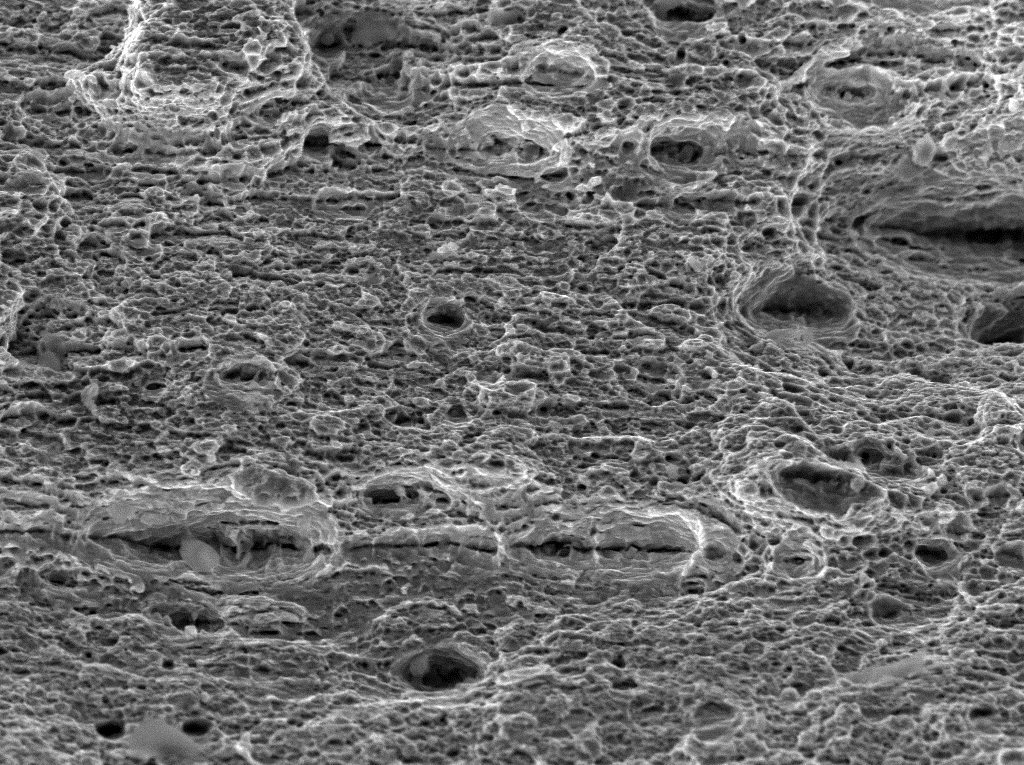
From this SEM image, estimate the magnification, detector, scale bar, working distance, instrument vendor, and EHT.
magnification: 1 K X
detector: SE Detector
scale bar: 50000 nm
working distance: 17.23 mm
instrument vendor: Tescan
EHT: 30 kV